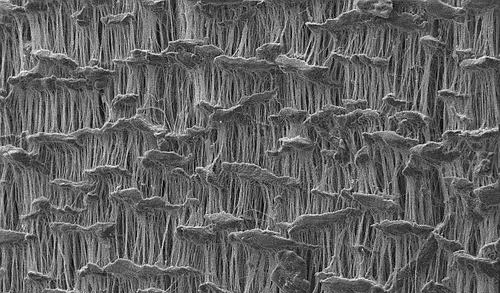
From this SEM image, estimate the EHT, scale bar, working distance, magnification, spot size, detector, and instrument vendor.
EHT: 10 kV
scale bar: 50000 nm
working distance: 10.5 mm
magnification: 1 K X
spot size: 3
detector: SE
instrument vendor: FEI